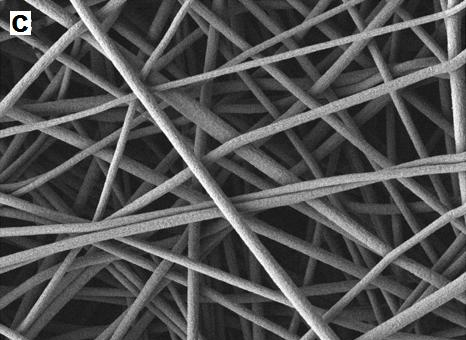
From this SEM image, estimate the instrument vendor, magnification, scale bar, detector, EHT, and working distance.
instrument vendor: JEOL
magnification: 5 K X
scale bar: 1000 nm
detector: LEI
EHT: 3 kV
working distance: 7 mm